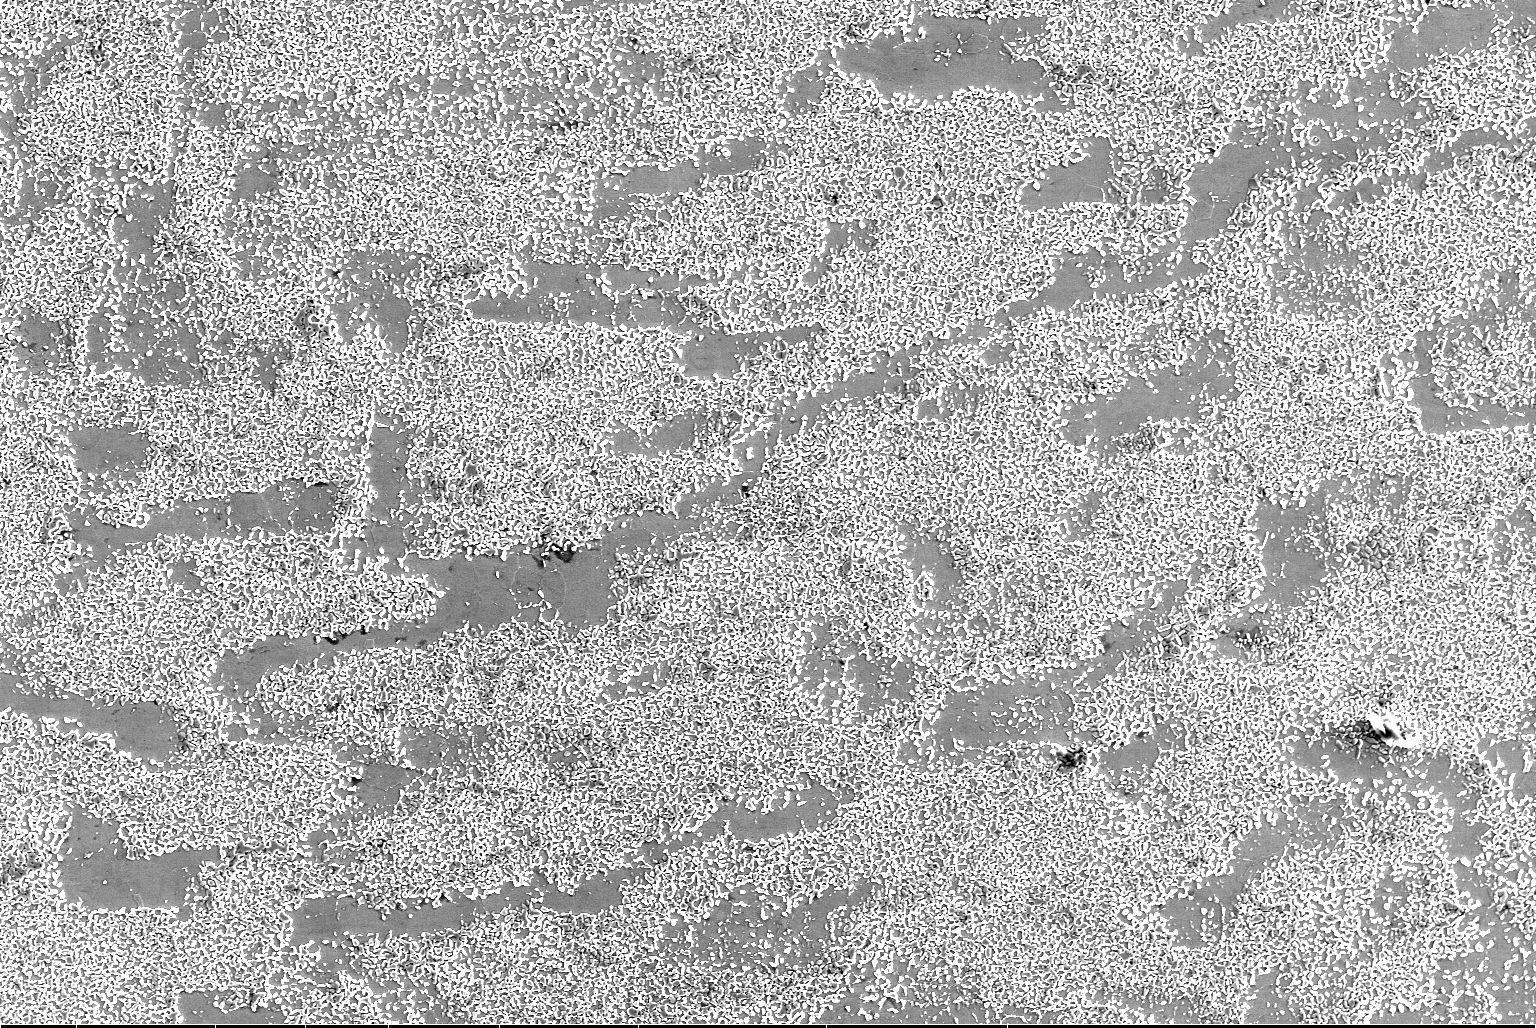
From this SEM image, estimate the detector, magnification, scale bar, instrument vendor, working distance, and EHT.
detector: ETD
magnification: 0.5 K X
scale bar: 200000 nm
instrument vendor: FEI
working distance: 13.2 mm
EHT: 20 kV